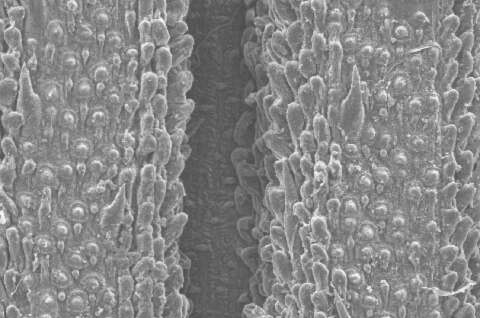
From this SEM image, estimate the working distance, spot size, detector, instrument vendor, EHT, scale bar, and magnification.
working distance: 10.1 mm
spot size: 2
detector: SE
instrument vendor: FEI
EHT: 10 kV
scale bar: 50000 nm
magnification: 0.4 K X